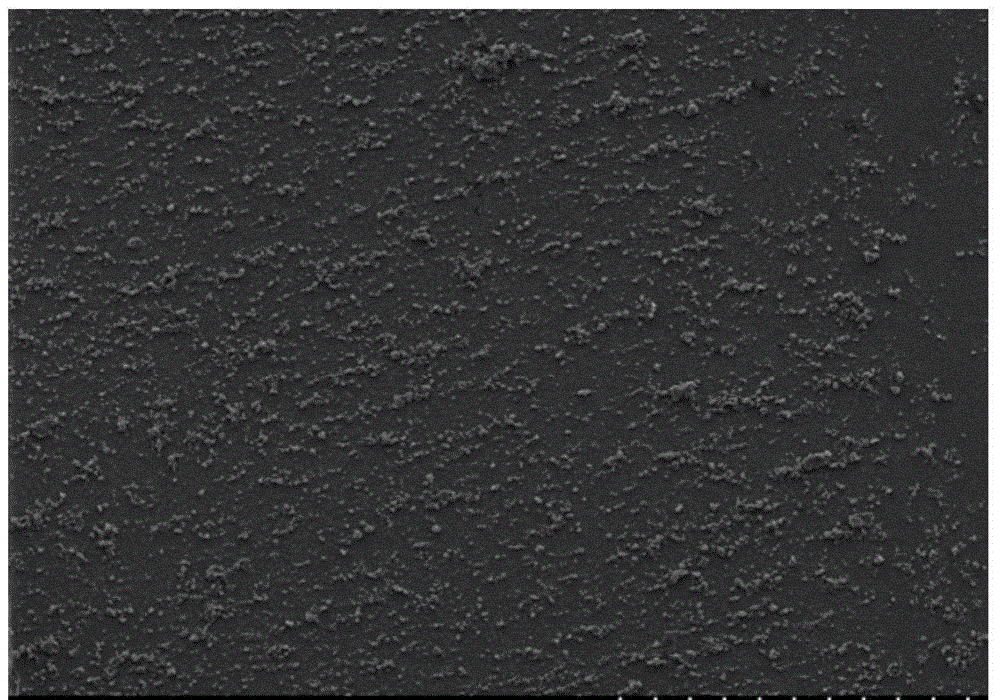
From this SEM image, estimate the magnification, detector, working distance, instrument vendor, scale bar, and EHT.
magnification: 0.045 K X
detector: SE(M)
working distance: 14.6 mm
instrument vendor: Hitachi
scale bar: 1000000 nm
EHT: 15 kV